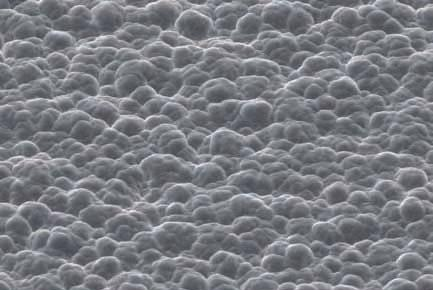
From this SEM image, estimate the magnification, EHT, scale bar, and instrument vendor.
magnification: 0.5 K X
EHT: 20 kV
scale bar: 50000 nm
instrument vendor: JEOL